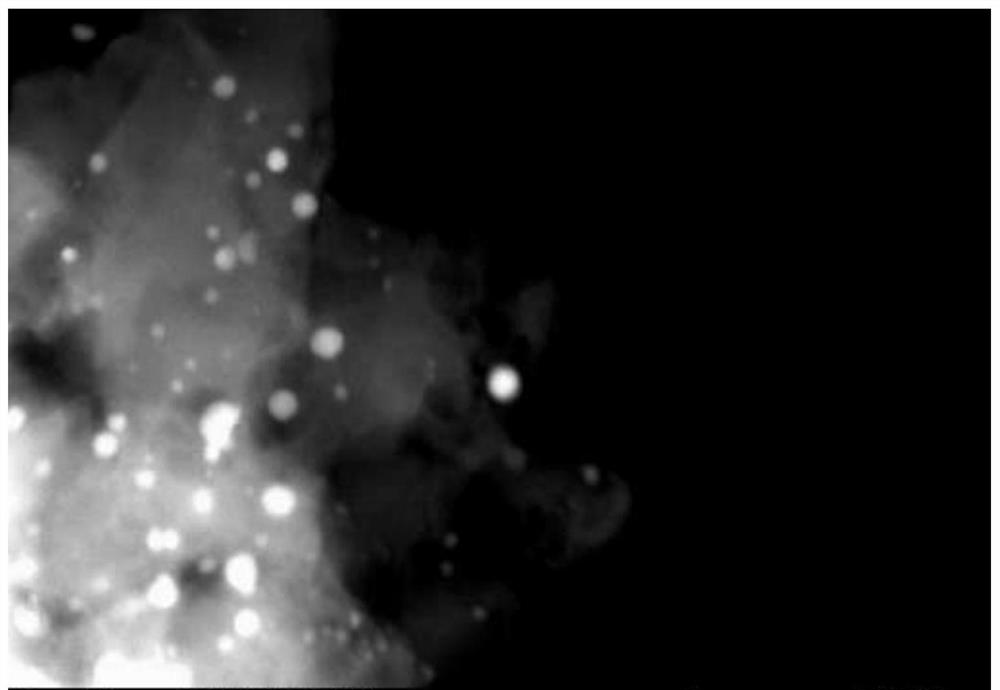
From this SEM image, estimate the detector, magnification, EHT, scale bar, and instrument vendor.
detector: STEM DF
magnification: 500 K X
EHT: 200 kV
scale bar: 50 nm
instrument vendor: JEOL